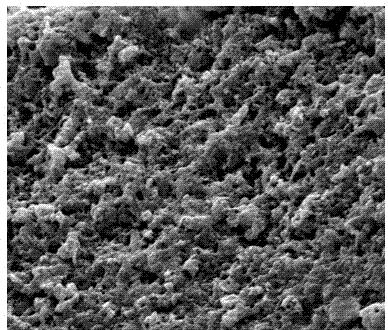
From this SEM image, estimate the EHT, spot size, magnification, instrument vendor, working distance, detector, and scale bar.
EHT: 5 kV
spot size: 3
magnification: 30 K X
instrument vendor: FEI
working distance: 10.1 mm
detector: ETD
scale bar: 3000 nm